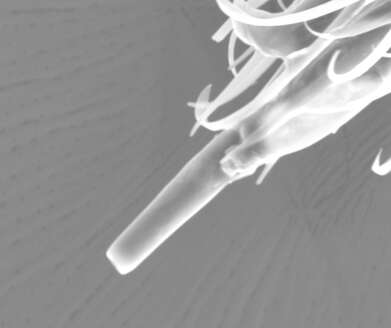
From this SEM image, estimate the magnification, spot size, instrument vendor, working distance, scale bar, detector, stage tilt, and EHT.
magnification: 1.564 K X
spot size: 4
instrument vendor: FEI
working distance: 18.3 mm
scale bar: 20000 nm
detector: ETD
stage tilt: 0.4°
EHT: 15 kV